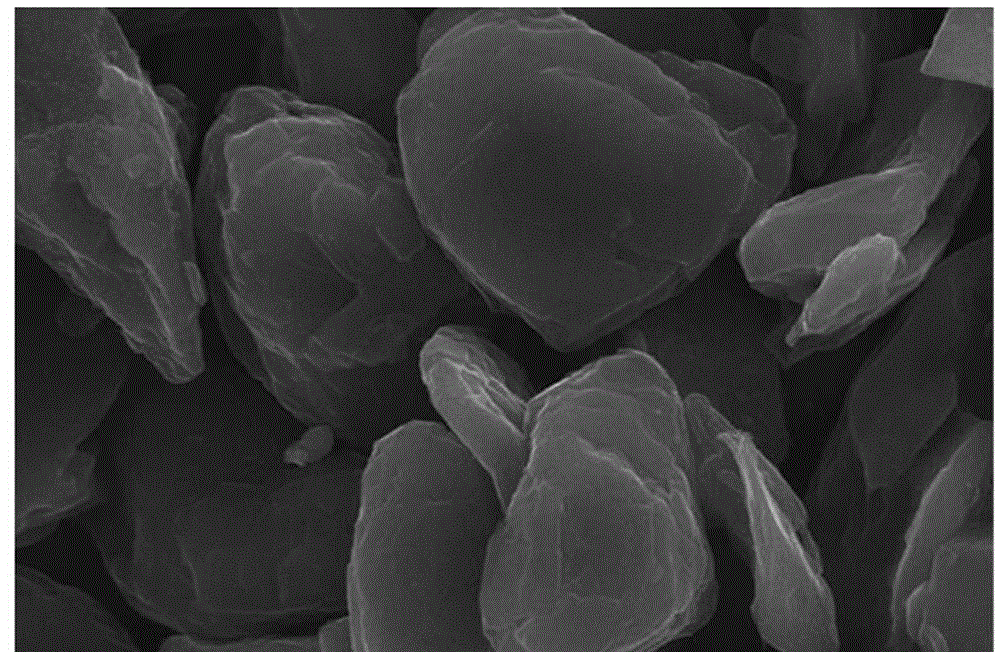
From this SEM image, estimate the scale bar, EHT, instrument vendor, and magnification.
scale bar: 5000 nm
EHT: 20 kV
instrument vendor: JEOL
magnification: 5 K X